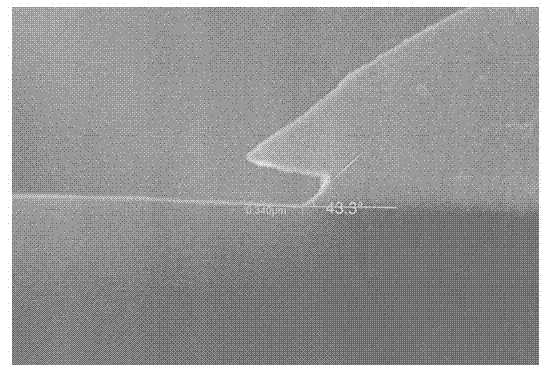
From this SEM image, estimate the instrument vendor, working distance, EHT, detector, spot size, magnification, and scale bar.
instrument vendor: JEOL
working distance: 10 mm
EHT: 20 kV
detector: SEI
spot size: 30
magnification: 40 K X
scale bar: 500 nm